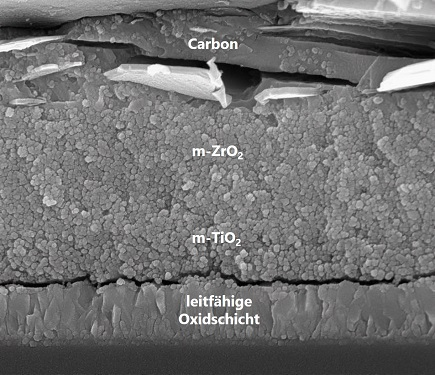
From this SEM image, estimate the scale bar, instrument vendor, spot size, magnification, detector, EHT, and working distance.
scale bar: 2000 nm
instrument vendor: FEI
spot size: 3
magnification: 66.237 K X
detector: TLD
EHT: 15 kV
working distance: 5.2 mm